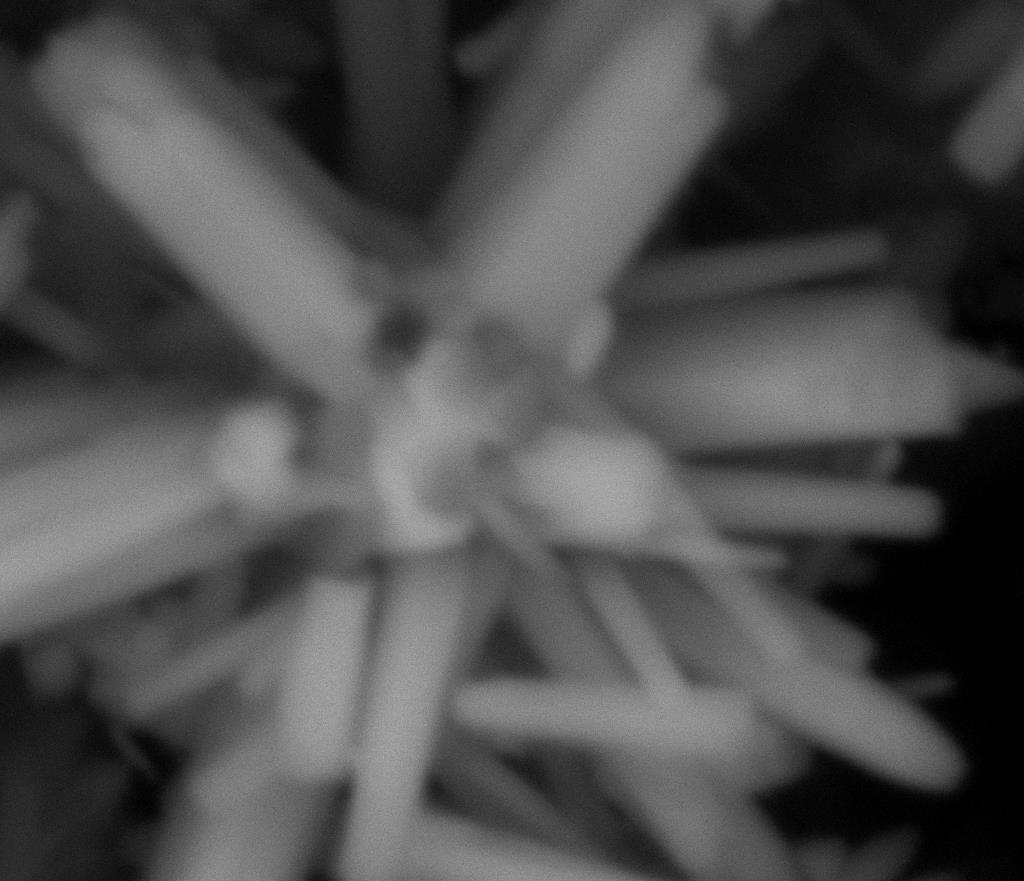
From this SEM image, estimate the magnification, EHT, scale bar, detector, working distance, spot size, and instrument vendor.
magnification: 12 K X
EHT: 25 kV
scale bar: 5000 nm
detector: Mix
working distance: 9.4 mm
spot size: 5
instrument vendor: FEI